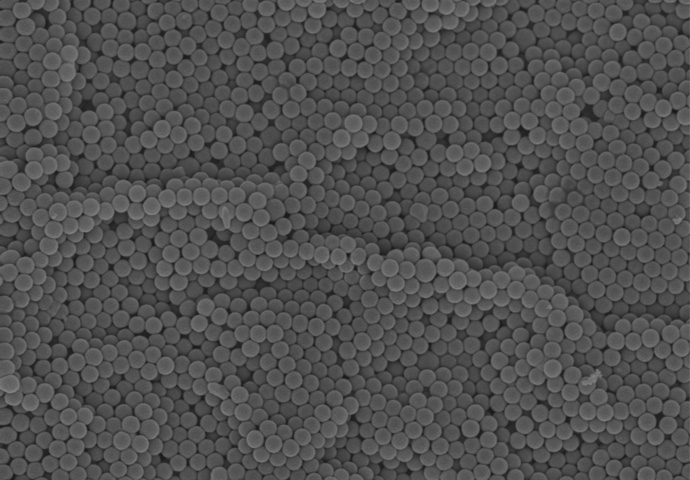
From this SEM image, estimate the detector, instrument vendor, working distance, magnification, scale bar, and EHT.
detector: InLens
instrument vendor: Zeiss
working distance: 6.5 mm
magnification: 50 K X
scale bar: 1000 nm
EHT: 5 kV